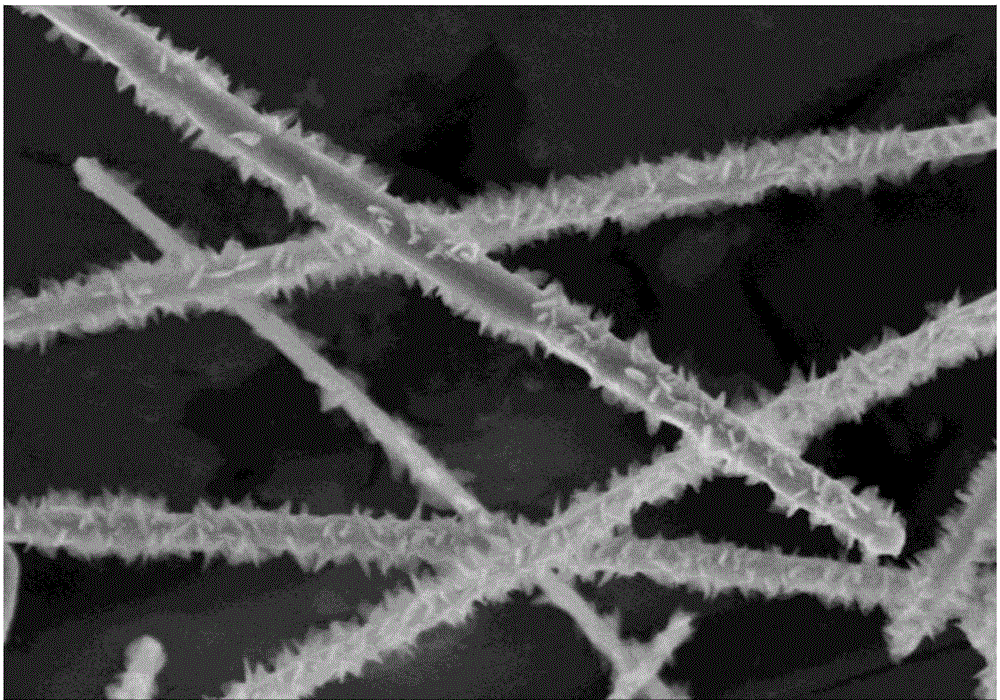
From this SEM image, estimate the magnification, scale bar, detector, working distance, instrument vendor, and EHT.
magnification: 40 K X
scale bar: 1000 nm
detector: SE(M)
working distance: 8 mm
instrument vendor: Hitachi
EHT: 5 kV